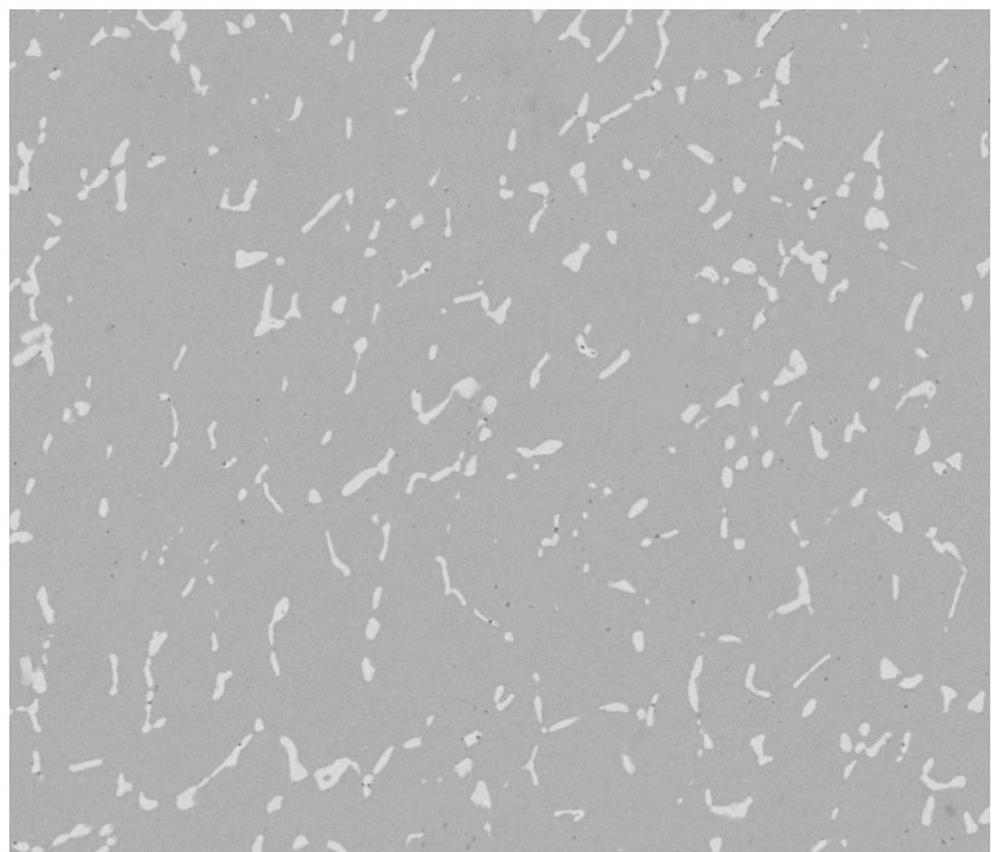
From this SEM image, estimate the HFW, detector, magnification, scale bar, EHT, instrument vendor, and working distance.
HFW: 149 µm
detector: BSED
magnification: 2 K X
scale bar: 50000 nm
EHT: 20 kV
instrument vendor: FEI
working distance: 11.8 mm